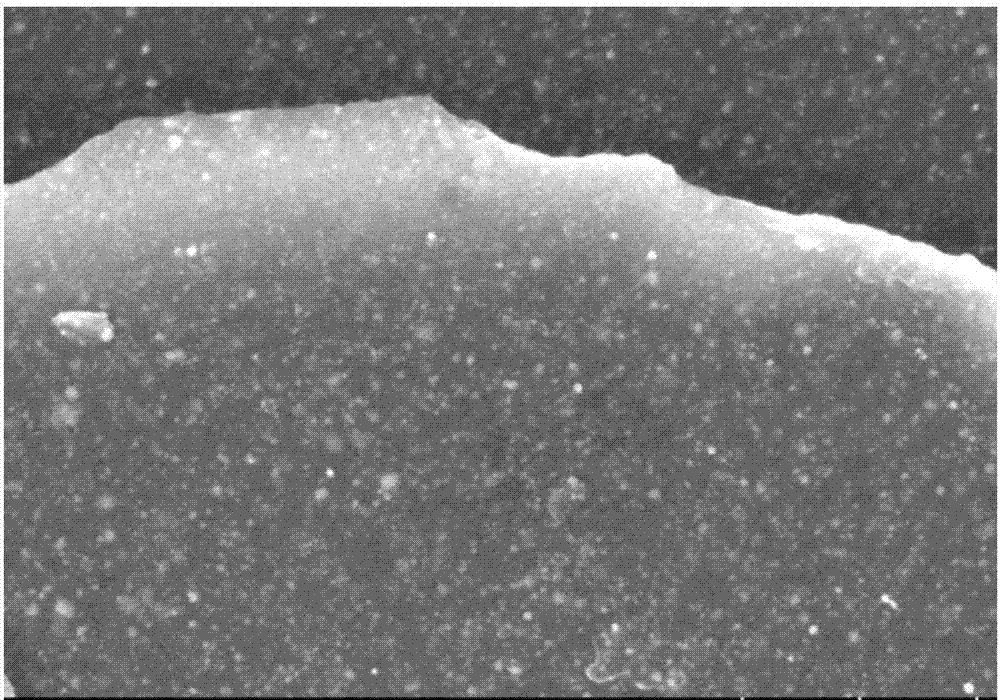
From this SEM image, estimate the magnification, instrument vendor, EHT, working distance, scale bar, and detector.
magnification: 60 K X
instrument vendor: Hitachi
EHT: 3 kV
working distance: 11.8 mm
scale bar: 500 nm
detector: SE(U)